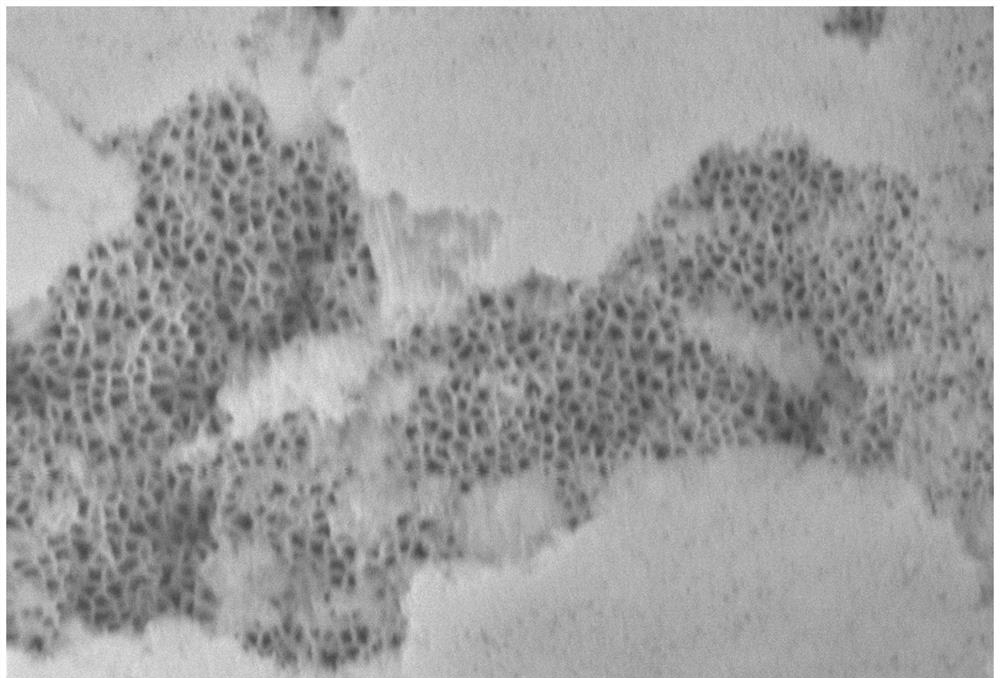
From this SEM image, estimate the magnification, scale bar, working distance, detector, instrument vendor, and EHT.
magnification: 50 K X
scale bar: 100 nm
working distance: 4.7 mm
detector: InLens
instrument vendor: Zeiss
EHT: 5 kV